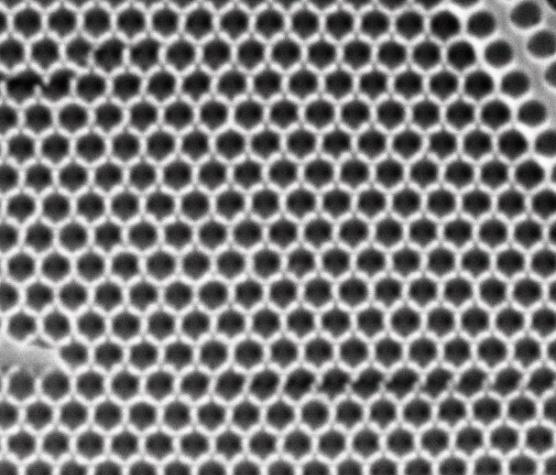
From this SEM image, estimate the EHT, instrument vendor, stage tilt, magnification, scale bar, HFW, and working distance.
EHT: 25 kV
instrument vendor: FEI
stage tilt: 0°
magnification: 50 K X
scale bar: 2000 nm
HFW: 5.41 µm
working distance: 10.6 mm